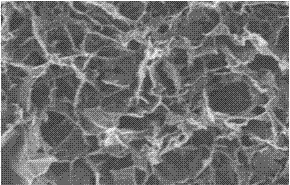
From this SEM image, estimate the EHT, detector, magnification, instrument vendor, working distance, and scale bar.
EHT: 5 kV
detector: SE(M)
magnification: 50 K X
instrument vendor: Hitachi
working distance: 8.4 mm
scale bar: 1000 nm